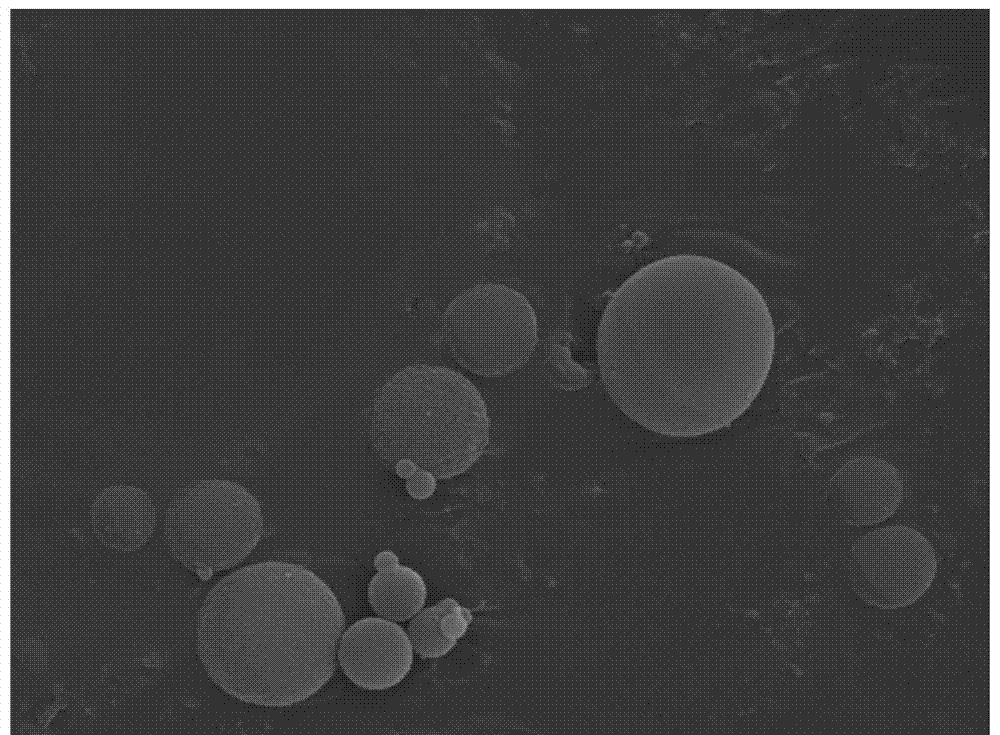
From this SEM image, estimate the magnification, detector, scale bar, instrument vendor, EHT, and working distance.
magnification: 10 K X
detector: LED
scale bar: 1000 nm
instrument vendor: JEOL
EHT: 15 kV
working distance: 10.1 mm